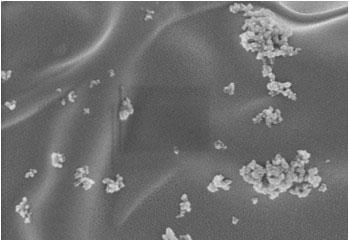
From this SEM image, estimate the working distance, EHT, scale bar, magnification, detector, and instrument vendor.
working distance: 7.8 mm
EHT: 2 kV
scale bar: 1000 nm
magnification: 50 K X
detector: SE(M)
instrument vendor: Hitachi